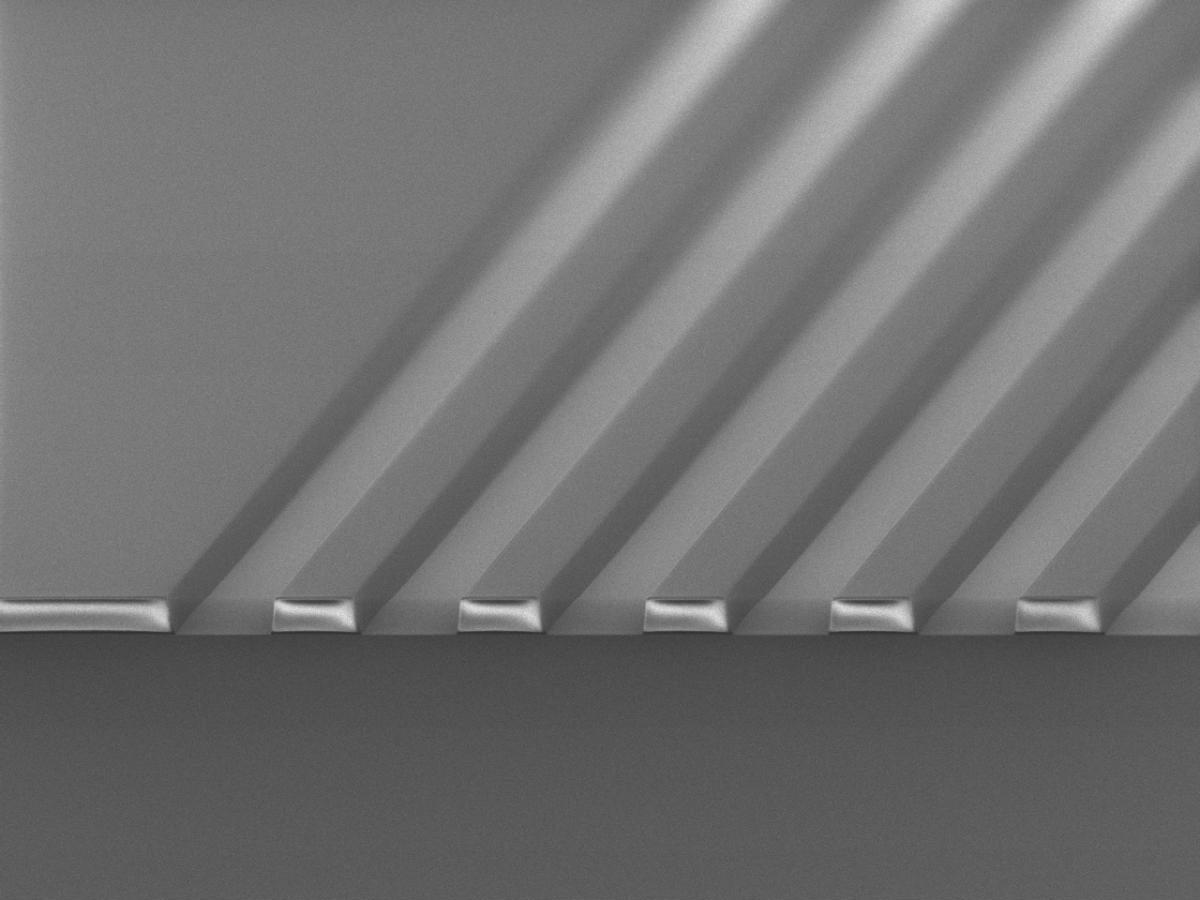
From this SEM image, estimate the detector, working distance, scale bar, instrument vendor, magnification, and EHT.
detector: SEI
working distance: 7.7 mm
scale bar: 10000 nm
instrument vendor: JEOL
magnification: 1 K X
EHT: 1.5 kV